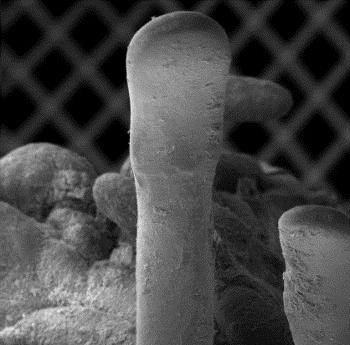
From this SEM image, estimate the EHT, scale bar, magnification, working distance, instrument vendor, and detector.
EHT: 15 kV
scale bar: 200000 nm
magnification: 0.056 K X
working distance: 8 mm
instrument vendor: other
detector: SE